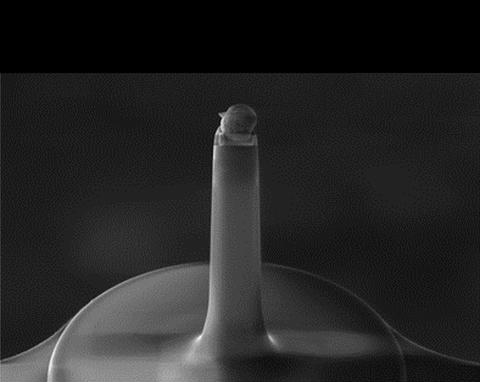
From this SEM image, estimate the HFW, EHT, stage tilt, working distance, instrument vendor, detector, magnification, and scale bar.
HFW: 23 µm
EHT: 5 kV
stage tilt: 52°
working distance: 4.3 mm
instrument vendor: FEI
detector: ICE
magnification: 6.5 K X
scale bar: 5000 nm